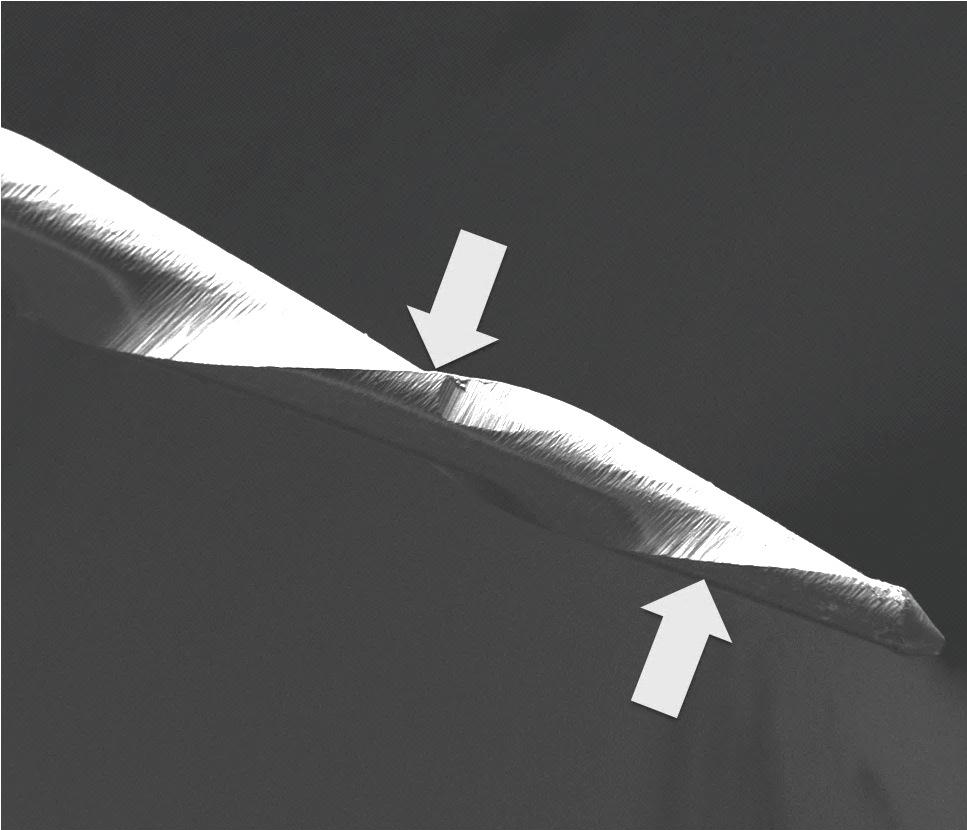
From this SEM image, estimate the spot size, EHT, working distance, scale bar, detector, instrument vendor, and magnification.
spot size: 6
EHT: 20 kV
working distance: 10.9 mm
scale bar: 1000000 nm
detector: ETD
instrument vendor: FEI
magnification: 0.15 K X